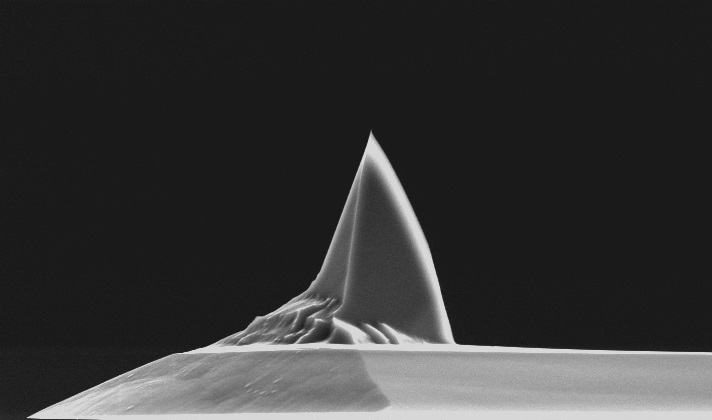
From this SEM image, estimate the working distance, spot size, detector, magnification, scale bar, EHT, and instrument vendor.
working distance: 22.3 mm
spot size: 3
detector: SE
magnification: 3 K X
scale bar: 10000 nm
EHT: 10 kV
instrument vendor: FEI